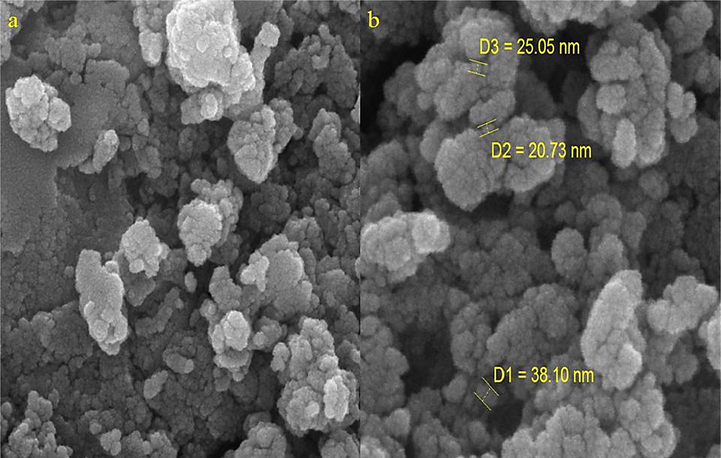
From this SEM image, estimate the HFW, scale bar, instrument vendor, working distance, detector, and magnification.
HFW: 2.08 µm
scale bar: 500 nm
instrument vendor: Tescan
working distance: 5 mm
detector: InBeam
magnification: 100 K X